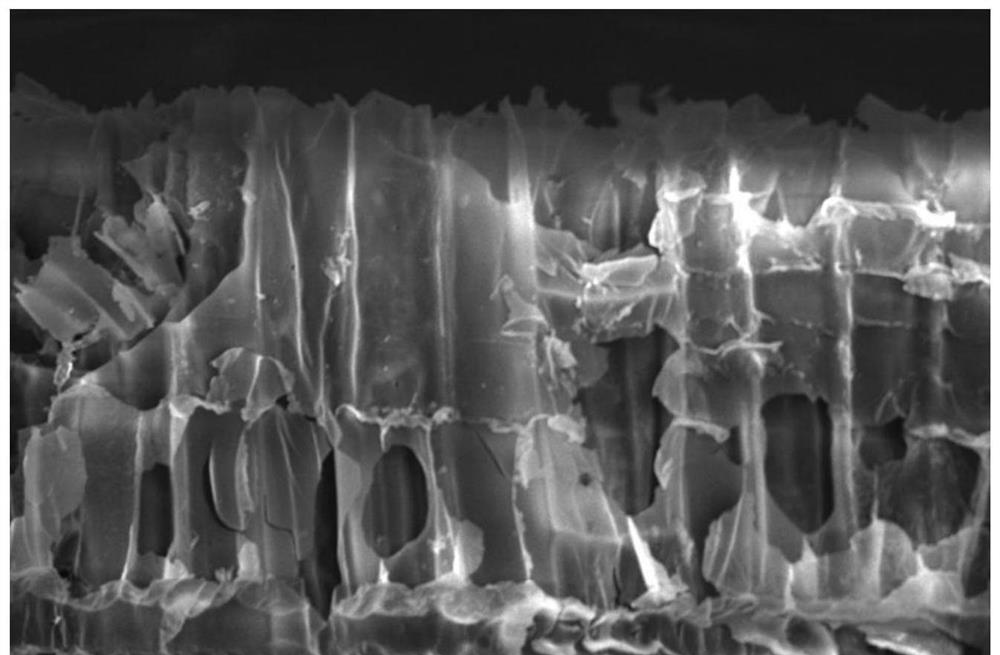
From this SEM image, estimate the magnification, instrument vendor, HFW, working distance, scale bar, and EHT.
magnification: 0.7 K X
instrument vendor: FEI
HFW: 296 µm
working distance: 10.1 mm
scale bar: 50000 nm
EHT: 15 kV